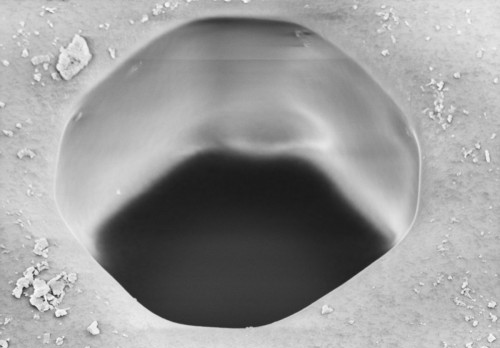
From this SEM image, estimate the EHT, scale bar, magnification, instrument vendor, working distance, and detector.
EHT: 5 kV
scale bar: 2000 nm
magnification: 10 K X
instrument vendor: Zeiss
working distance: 15.5 mm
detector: SE2